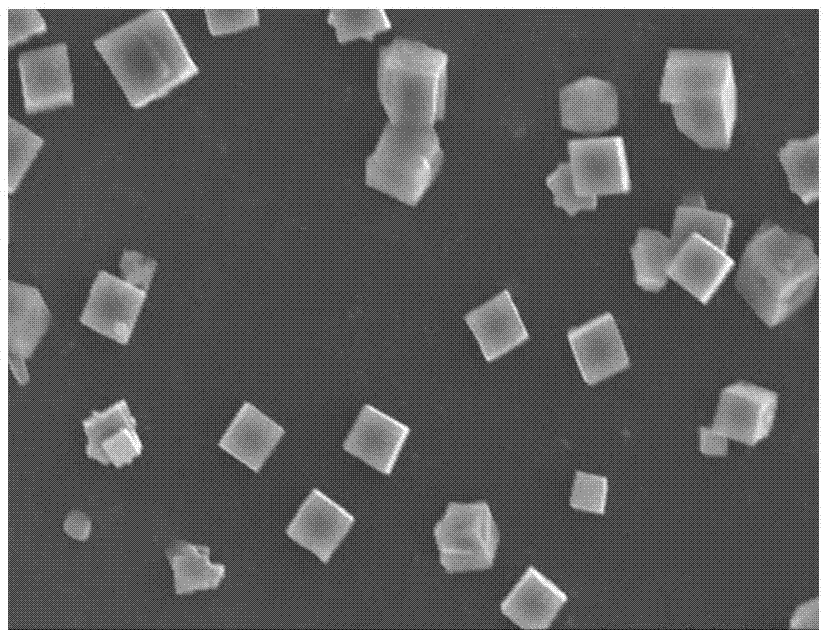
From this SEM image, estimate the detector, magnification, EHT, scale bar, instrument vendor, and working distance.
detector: SE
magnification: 1.5 K X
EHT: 15 kV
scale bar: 30000 nm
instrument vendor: Hitachi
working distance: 11 mm